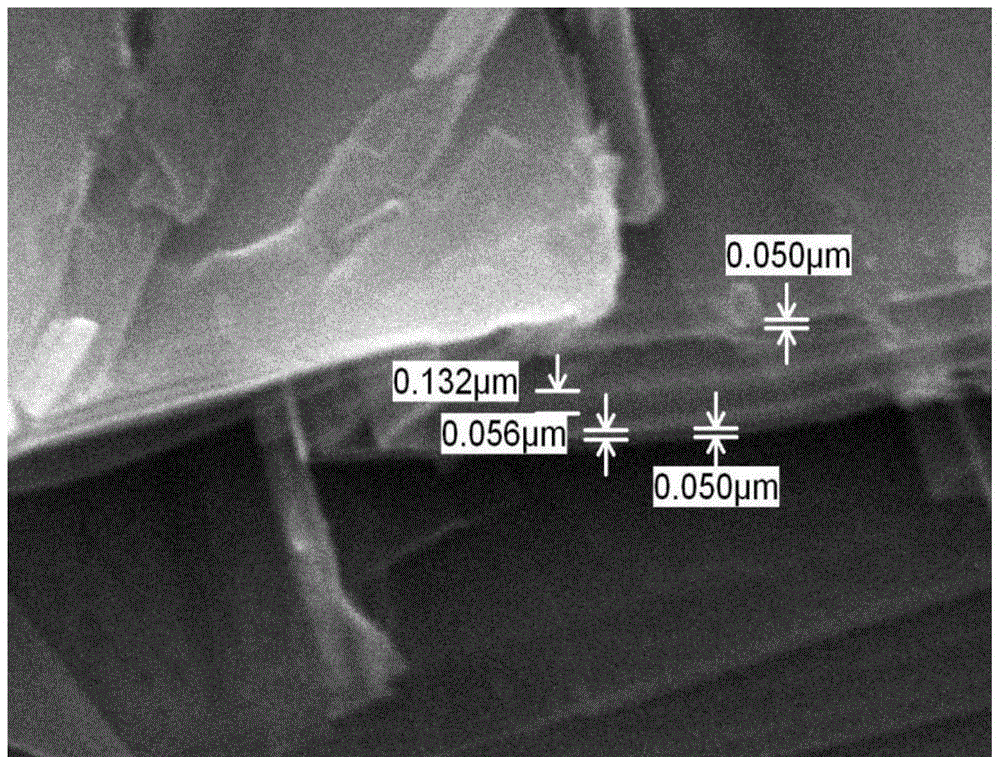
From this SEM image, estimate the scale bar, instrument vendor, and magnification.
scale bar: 1000 nm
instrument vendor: JEOL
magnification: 20 K X